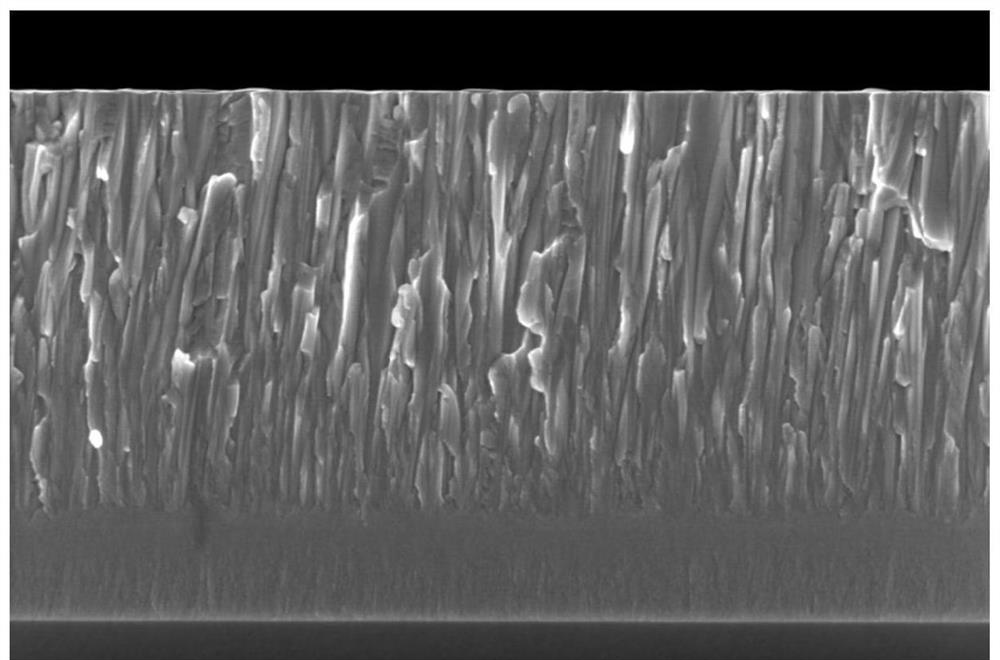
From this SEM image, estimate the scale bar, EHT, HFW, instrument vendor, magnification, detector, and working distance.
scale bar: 1000 nm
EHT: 5 kV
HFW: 4.14 µm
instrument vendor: FEI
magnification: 50 K X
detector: TLD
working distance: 4.4 mm